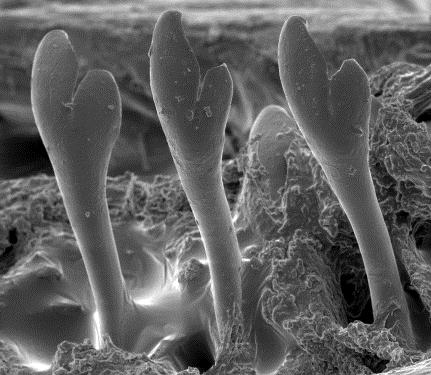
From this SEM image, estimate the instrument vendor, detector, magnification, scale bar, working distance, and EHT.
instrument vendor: other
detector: SE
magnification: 0.23 K X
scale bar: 50000 nm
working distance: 13 mm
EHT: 10 kV